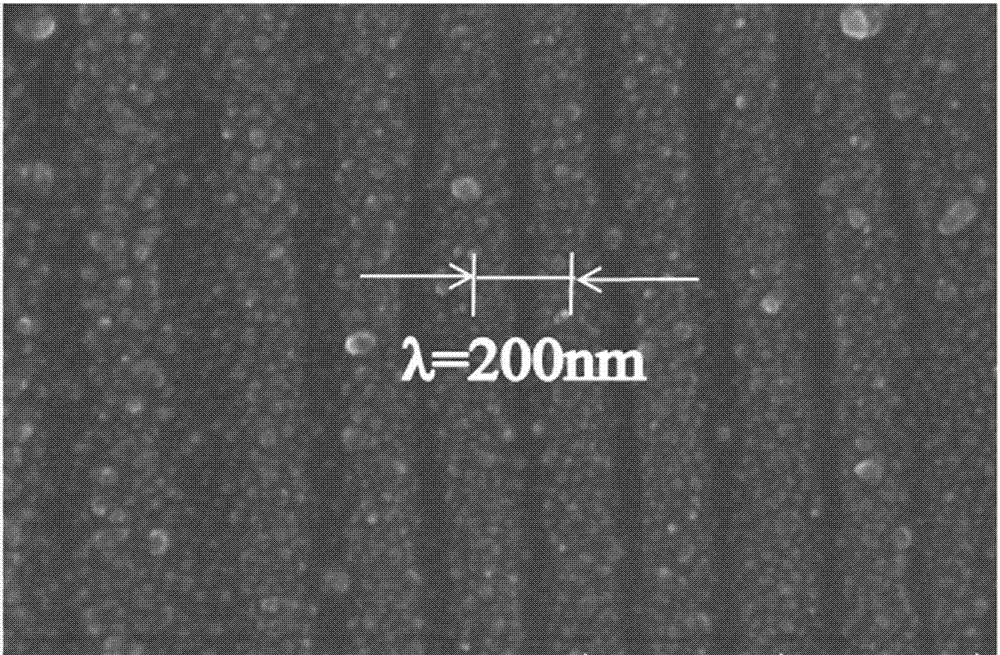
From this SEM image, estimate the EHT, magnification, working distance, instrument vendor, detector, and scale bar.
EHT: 11 kV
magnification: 50 K X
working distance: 16.7 mm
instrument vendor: Hitachi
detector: SE(UL)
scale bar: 1000 nm